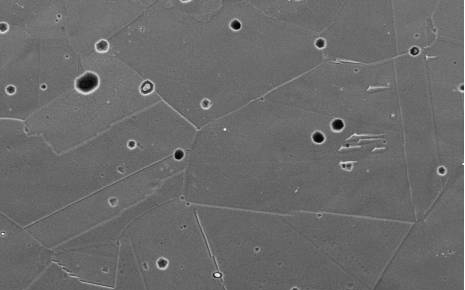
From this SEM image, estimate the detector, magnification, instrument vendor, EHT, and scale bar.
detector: SEI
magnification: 1 K X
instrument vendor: JEOL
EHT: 15 kV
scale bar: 20000 nm